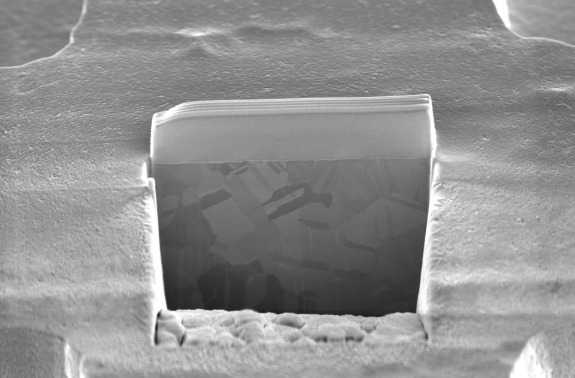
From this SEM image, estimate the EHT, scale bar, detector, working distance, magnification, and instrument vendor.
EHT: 5 kV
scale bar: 10000 nm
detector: TLD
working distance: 3.9 mm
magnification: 10 K X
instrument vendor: FEI